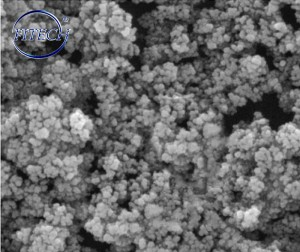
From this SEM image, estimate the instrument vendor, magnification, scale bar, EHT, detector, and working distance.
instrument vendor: Tescan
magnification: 75 K X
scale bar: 500 nm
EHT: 20 kV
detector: SE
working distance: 8 mm